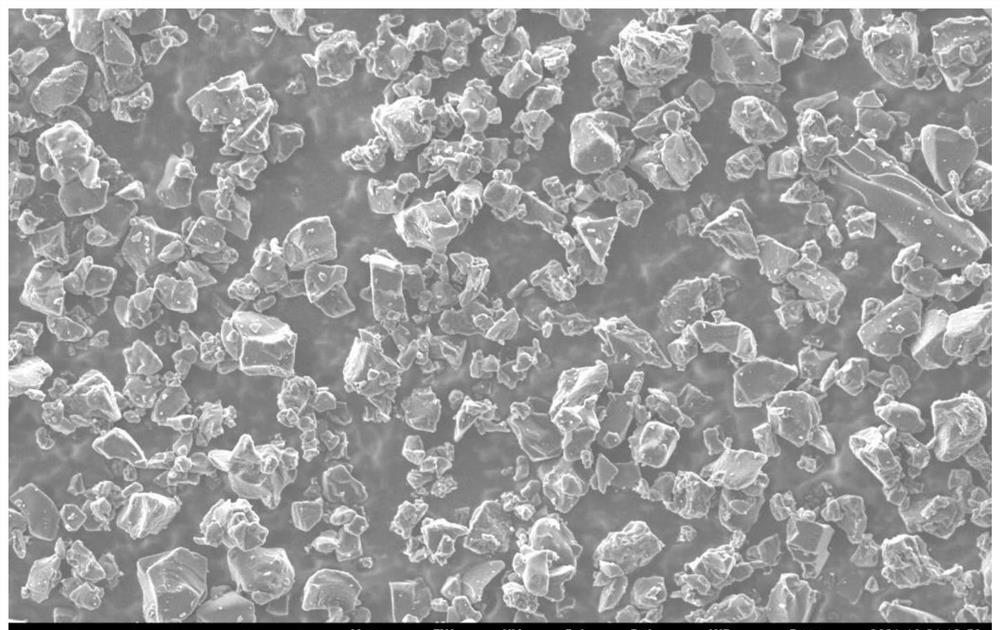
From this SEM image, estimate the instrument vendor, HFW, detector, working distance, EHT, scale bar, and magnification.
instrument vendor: other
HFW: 173 µm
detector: SED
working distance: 4.8 mm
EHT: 5 kV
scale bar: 50000 nm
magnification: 3 K X